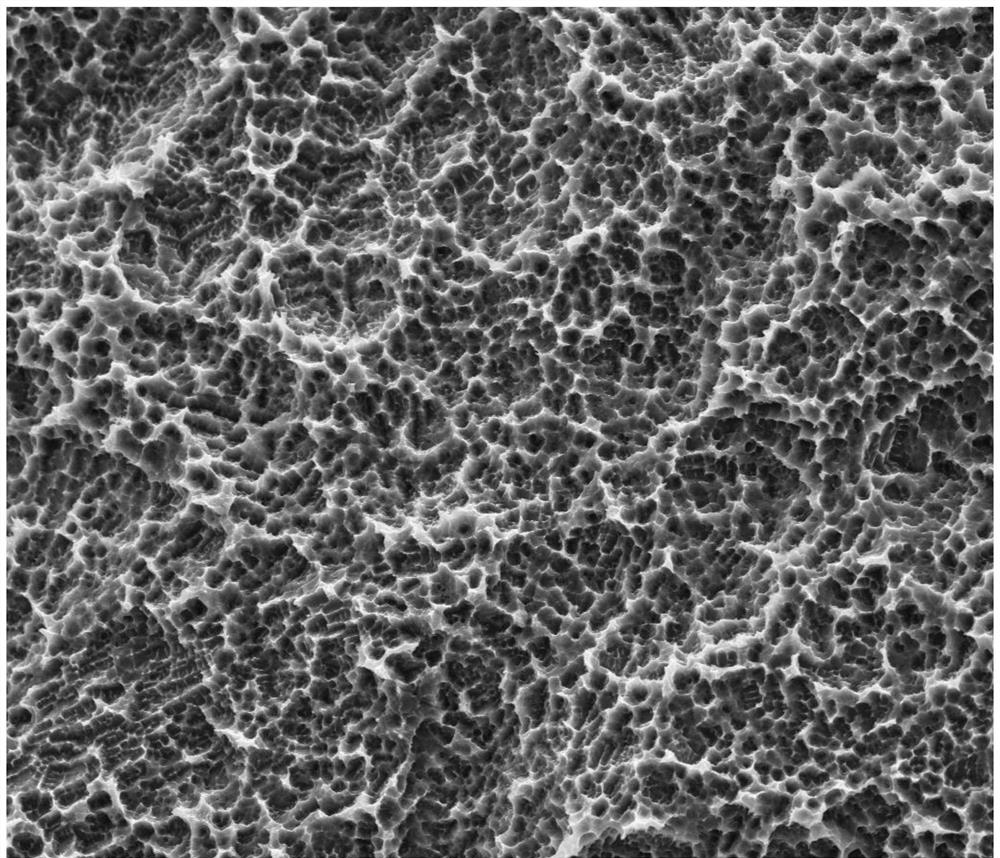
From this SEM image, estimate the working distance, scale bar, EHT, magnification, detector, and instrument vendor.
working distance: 6.9 mm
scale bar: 40000 nm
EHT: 20 kV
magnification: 3 K X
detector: ETD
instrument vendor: FEI